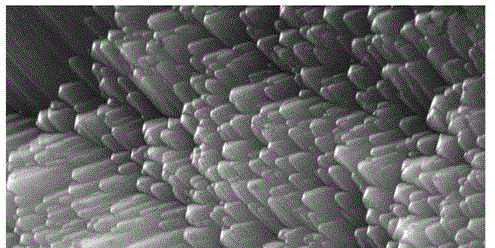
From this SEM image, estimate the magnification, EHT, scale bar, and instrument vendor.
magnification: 3 K X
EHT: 30 kV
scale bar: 5000 nm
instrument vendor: JEOL